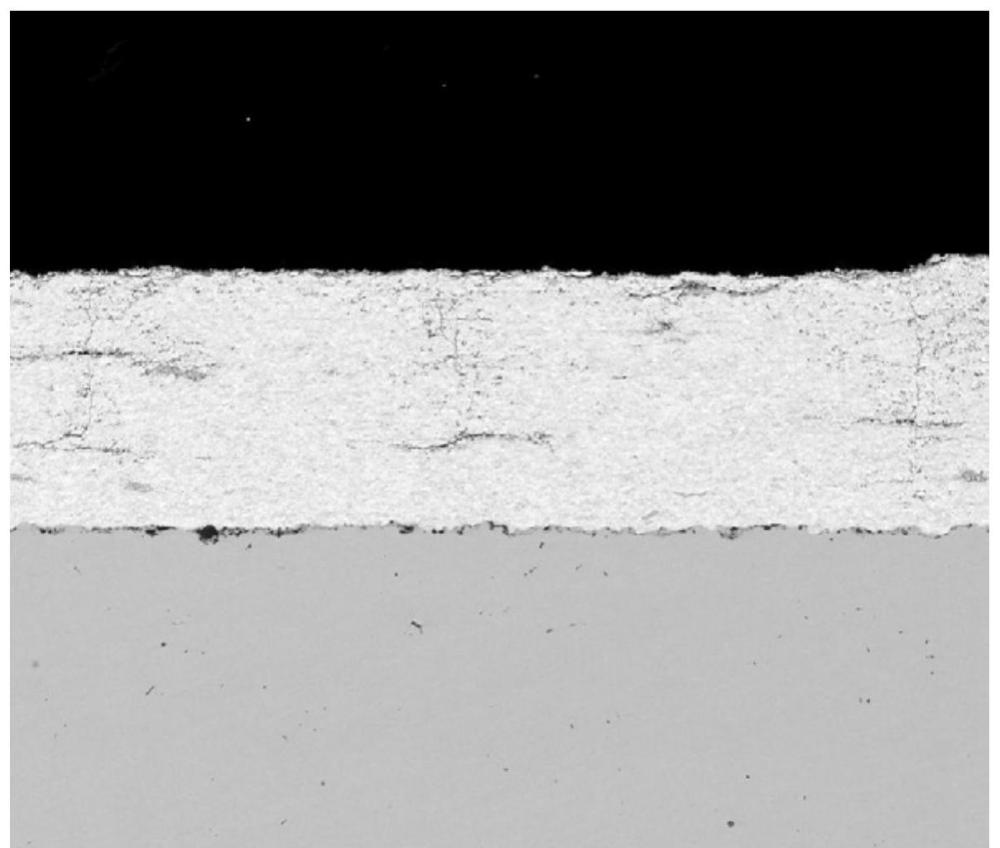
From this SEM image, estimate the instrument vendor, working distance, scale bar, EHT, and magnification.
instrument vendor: FEI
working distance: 10.7 mm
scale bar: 200000 nm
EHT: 20 kV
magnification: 0.5 K X